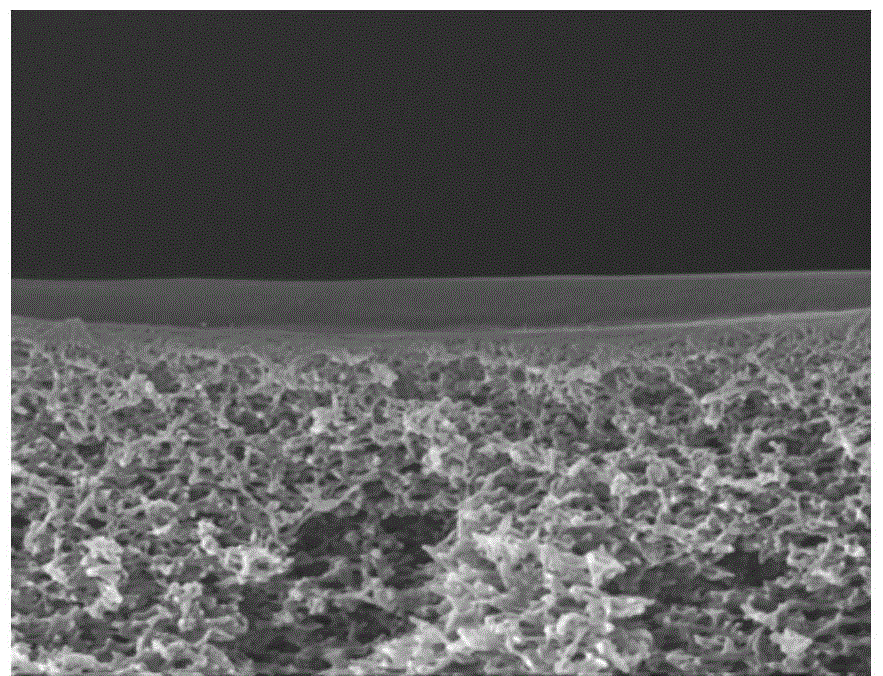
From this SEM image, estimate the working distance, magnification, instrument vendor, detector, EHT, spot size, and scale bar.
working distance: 4.4 mm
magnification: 50 K X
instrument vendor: FEI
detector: TLD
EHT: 10 kV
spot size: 3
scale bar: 1000 nm